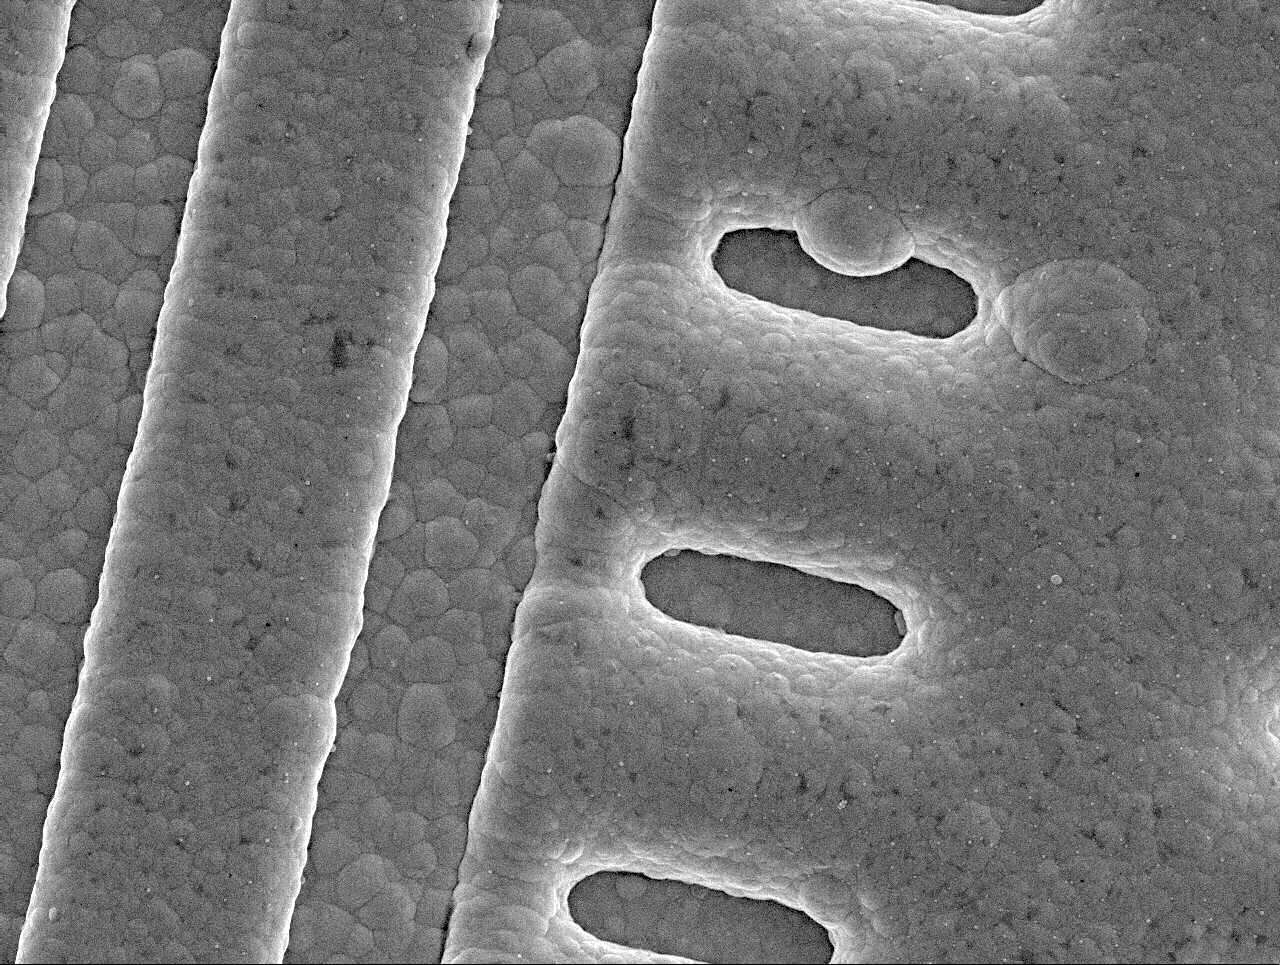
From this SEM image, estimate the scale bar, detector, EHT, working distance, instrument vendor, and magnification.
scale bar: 1000 nm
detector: SEI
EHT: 5 kV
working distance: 10 mm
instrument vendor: JEOL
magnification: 5 K X